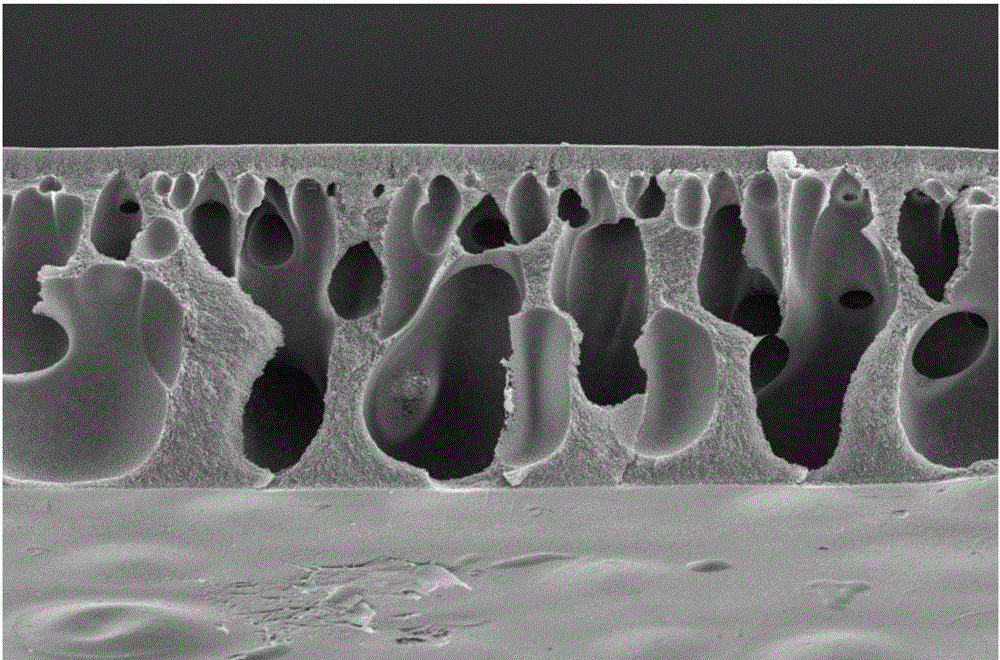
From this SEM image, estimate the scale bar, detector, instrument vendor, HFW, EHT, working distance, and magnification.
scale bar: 50000 nm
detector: ETD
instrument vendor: FEI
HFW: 423 µm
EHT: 10 kV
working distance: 13.5 mm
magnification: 0.3 K X